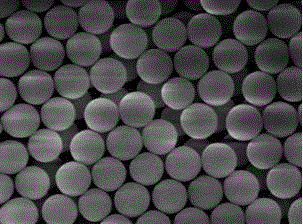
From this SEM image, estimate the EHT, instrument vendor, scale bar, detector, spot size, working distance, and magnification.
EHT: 5 kV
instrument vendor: FEI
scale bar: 500 nm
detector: TLD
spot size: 3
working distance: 4.9 mm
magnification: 40 K X